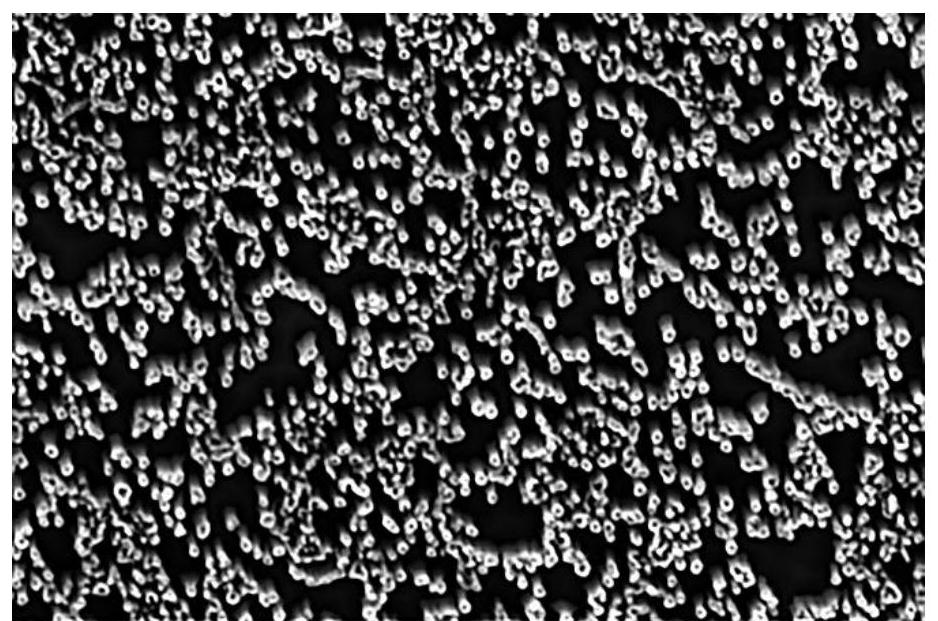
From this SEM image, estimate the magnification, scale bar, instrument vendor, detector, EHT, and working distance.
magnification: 10 K X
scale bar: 10000 nm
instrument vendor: FEI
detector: ETD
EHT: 10 kV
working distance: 9.7 mm